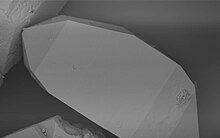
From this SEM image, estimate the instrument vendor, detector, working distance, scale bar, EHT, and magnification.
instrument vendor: Zeiss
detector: QBSD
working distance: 11 mm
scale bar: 10000 nm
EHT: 15 kV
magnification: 1.26 K X